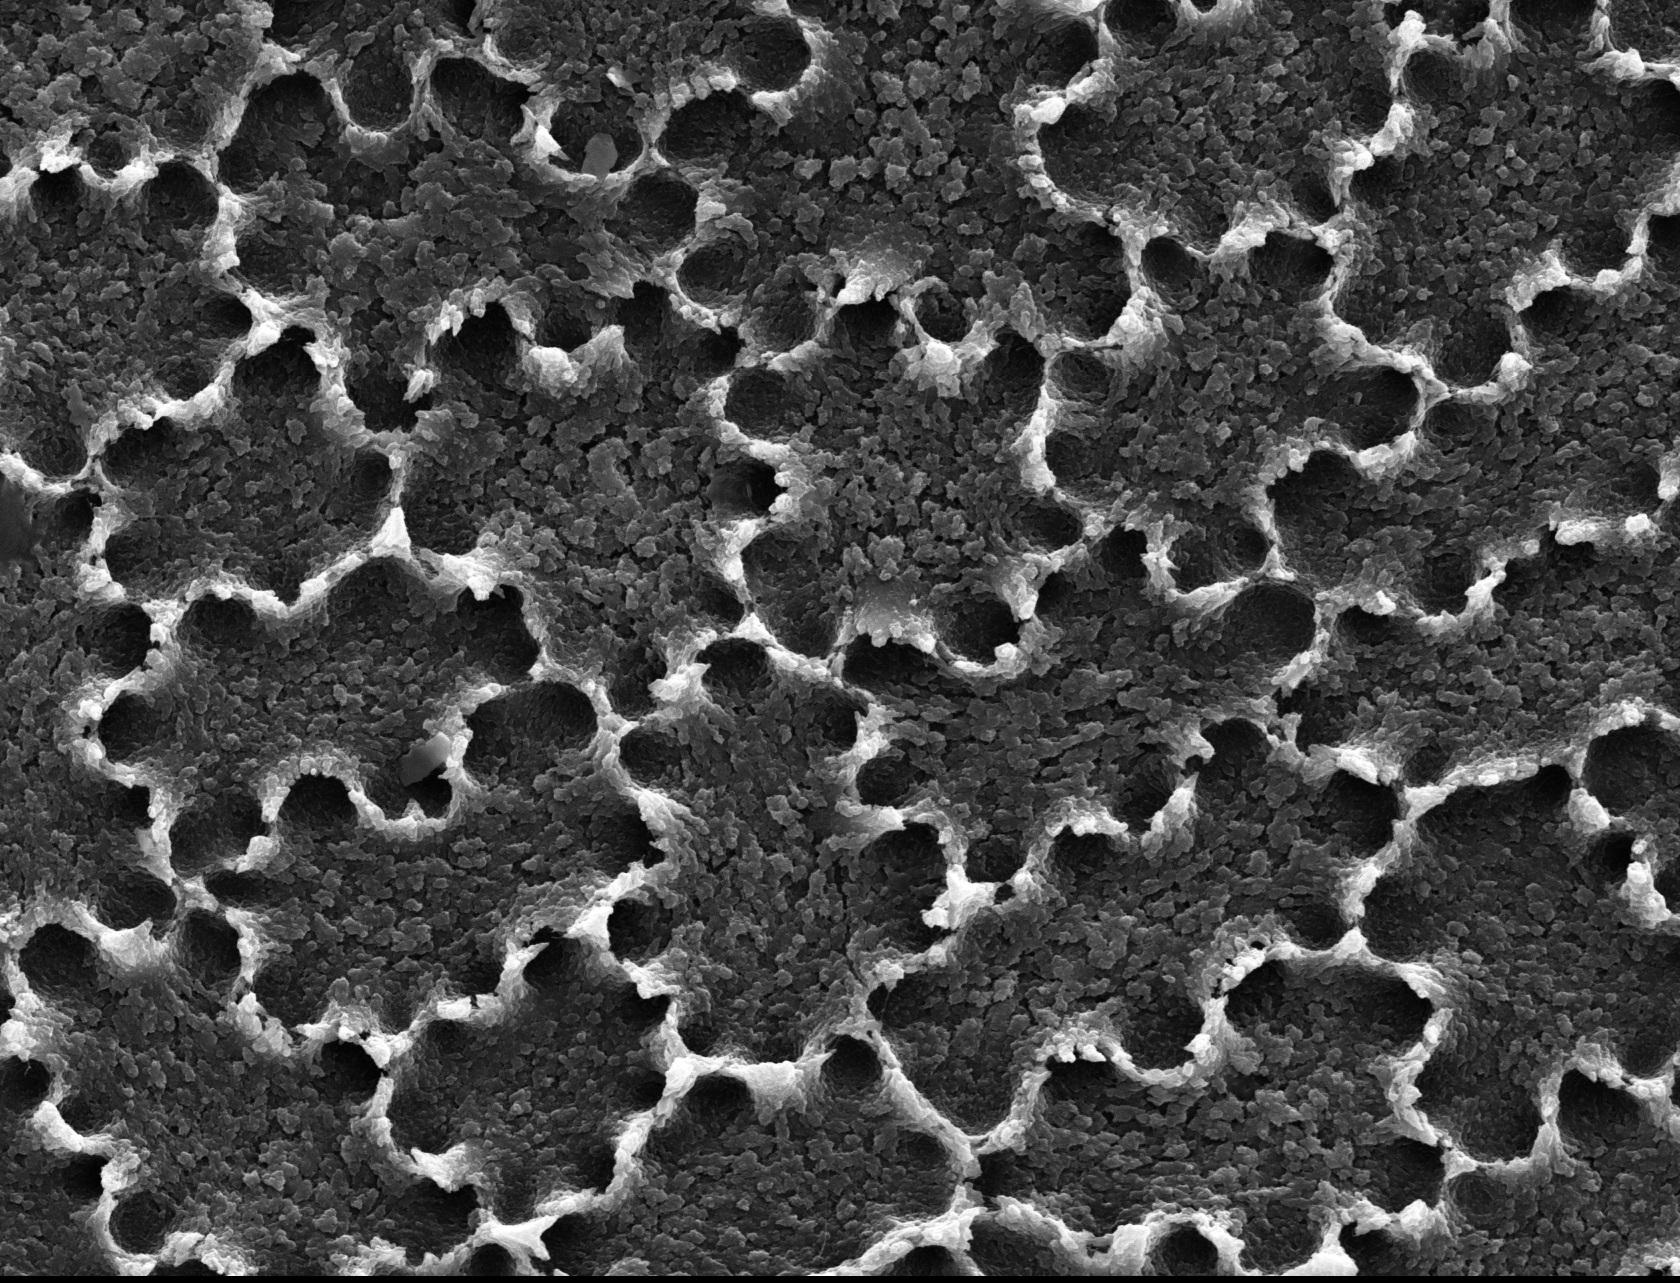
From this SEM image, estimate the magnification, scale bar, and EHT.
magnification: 0.829 K X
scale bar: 30000 nm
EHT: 15 kV